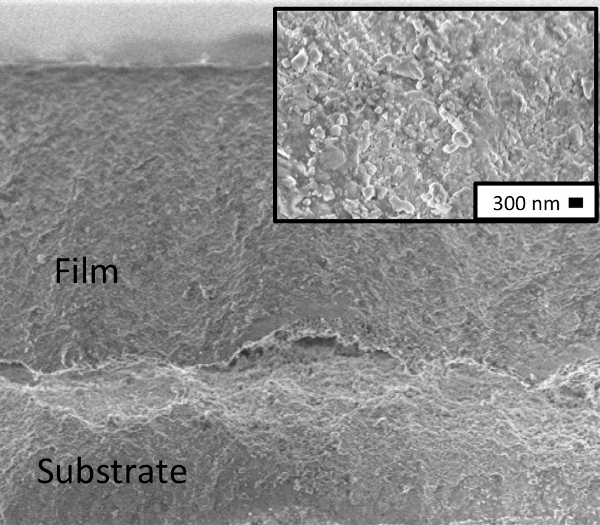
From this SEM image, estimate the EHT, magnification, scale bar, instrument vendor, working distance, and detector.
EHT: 3 kV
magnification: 2.15 K X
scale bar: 2000 nm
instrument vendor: Zeiss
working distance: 3.5 mm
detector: InLens